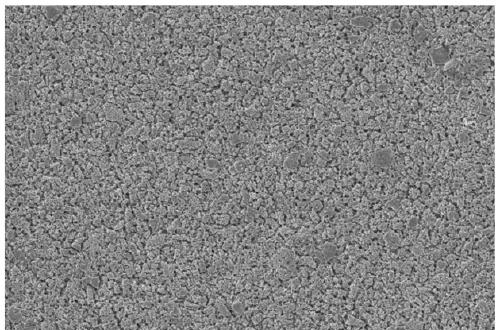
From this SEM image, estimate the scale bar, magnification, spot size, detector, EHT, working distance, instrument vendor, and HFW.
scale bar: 100000 nm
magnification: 1.5 K X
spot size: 2.5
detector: ETD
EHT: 10 kV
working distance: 19.4 mm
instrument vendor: FEI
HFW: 276 µm